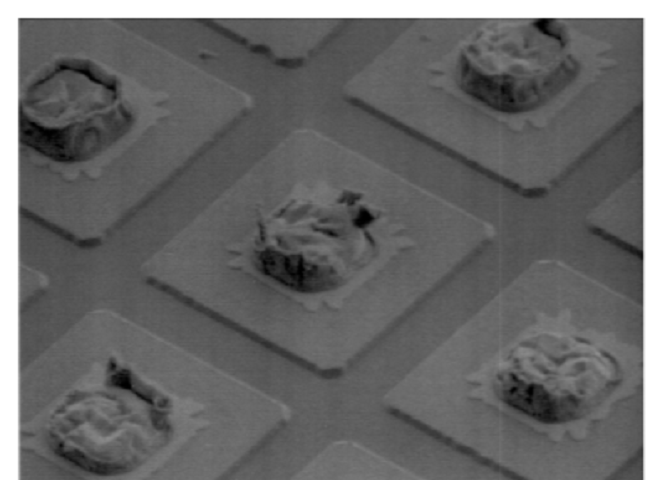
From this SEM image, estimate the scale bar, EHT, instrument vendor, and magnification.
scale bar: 15000 nm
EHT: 4 kV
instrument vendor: Hitachi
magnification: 2 K X